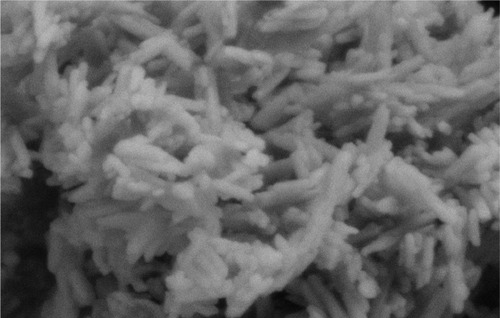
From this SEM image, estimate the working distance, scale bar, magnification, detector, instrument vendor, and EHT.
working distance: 3.4 mm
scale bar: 100 nm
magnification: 100 K X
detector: SE2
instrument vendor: Zeiss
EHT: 2 kV